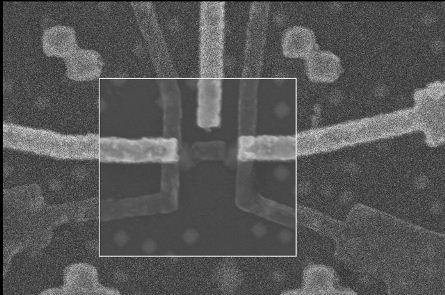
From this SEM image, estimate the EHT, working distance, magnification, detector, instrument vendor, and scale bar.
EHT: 5 kV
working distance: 5.8 mm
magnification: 61.63 K X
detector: InLens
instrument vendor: Zeiss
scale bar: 200 nm